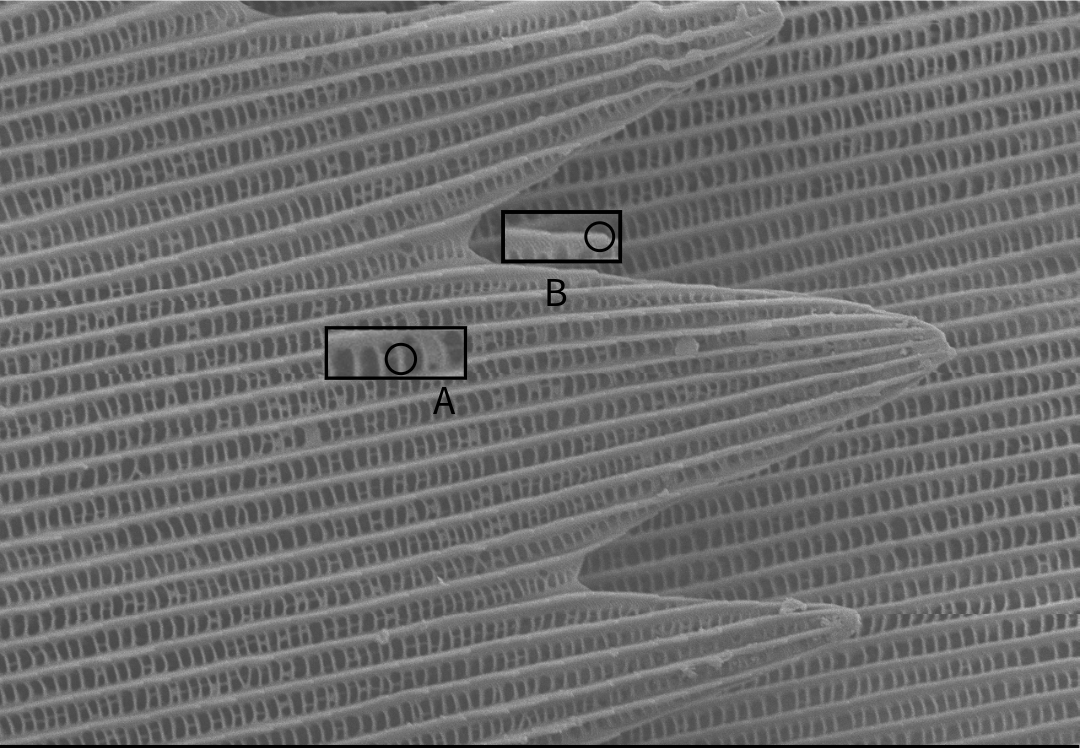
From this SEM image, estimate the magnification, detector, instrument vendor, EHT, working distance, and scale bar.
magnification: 2 K X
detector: SEI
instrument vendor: JEOL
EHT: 5 kV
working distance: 13.3 mm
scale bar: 10000 nm